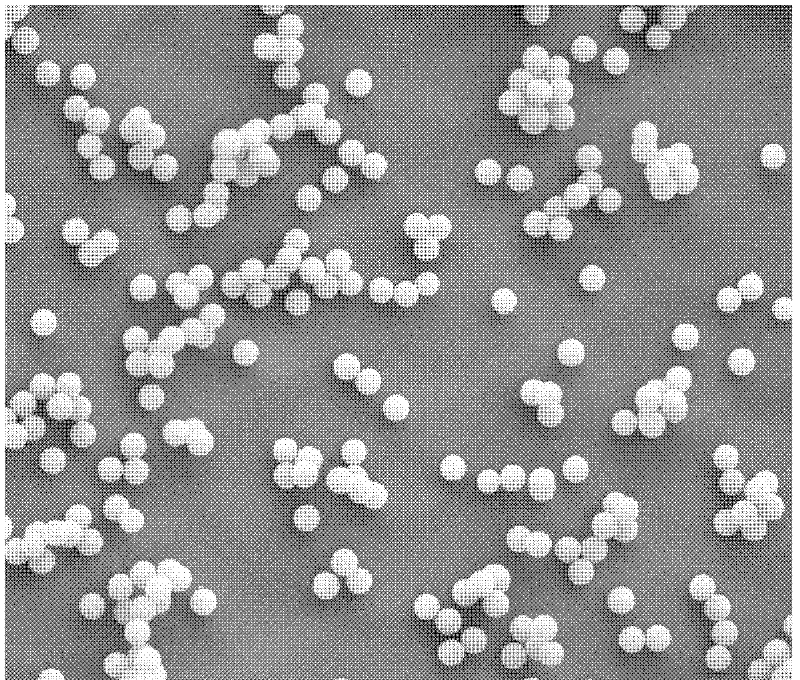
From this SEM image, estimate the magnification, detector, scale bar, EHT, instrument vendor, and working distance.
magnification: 3 K X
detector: ETD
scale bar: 40000 nm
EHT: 10 kV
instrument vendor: FEI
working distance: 7 mm